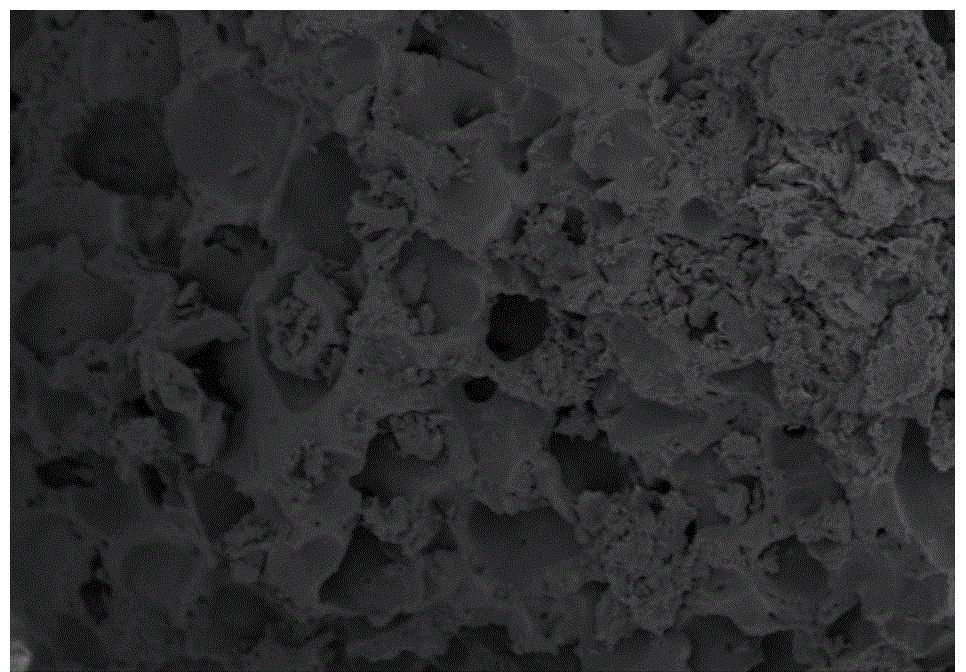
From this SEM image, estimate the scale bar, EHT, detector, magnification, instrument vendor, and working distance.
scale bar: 100000 nm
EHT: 5 kV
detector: SE(M)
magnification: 0.5 K X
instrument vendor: Hitachi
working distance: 8.5 mm